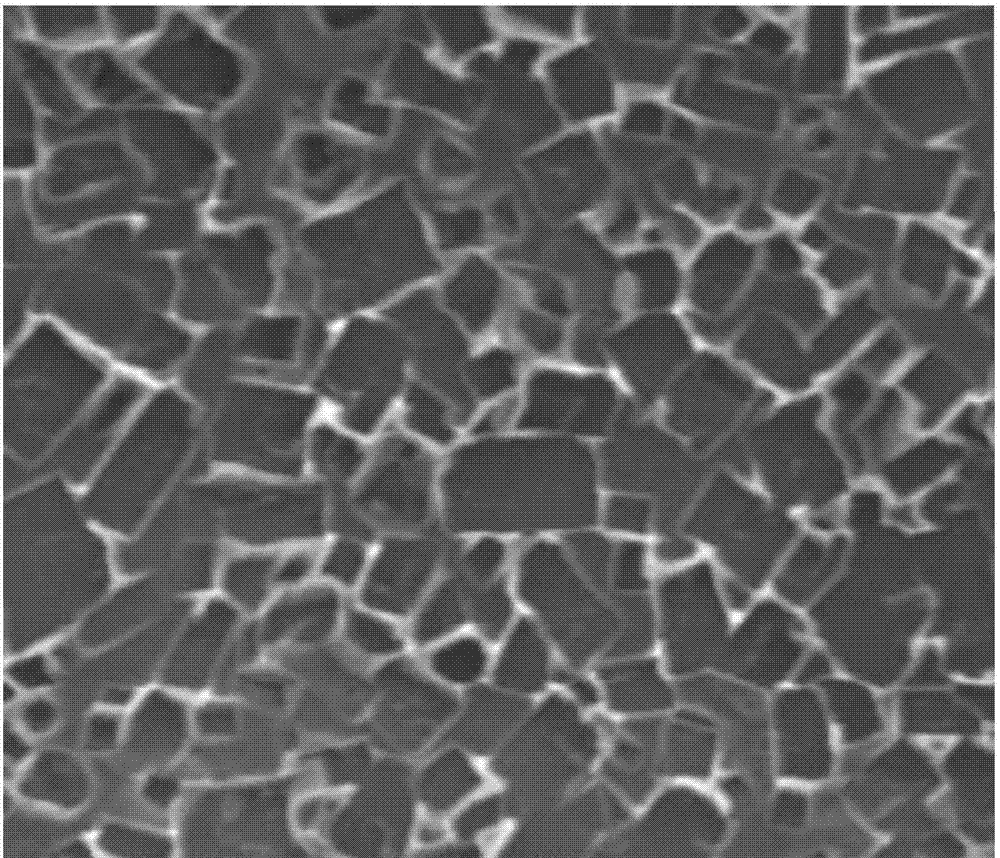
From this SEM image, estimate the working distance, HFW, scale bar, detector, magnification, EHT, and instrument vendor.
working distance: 10.8 mm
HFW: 10.82 µm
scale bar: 2000 nm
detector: ETD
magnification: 25 K X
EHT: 20 kV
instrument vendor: FEI